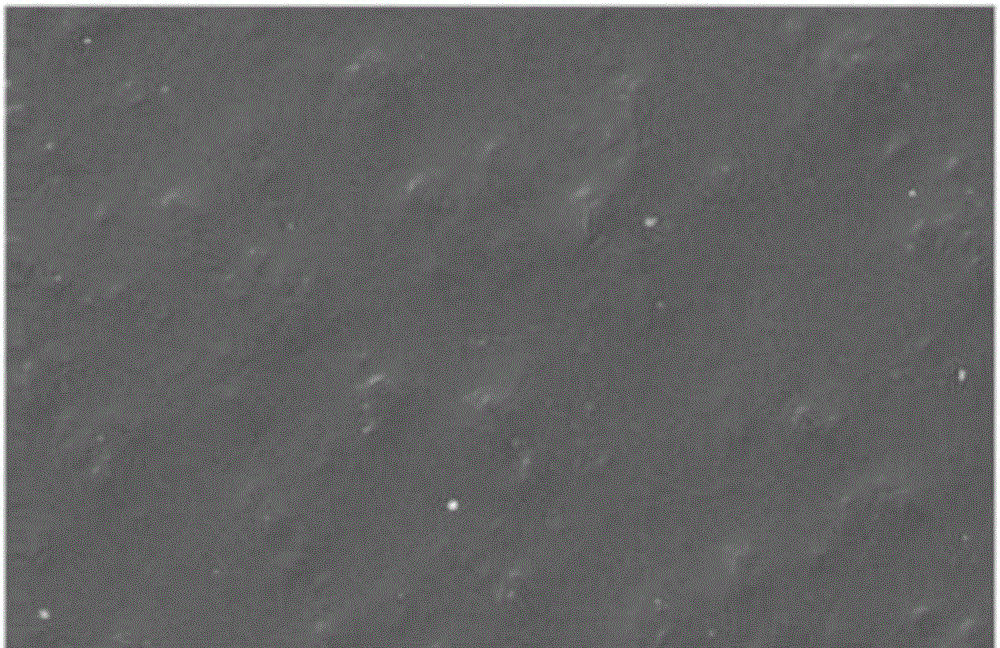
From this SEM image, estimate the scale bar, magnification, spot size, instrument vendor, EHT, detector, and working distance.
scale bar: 20000 nm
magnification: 1 K X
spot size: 3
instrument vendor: FEI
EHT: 20 kV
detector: SE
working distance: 19.9 mm